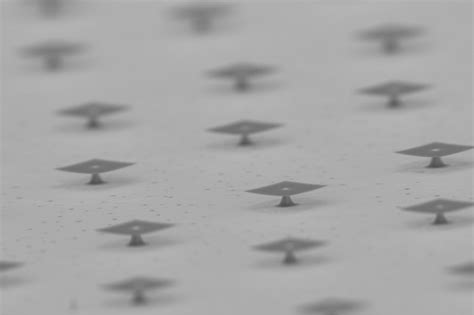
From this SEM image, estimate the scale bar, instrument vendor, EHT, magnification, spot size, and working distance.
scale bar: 10000 nm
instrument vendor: JEOL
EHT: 10 kV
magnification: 1 K X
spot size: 50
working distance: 9 mm